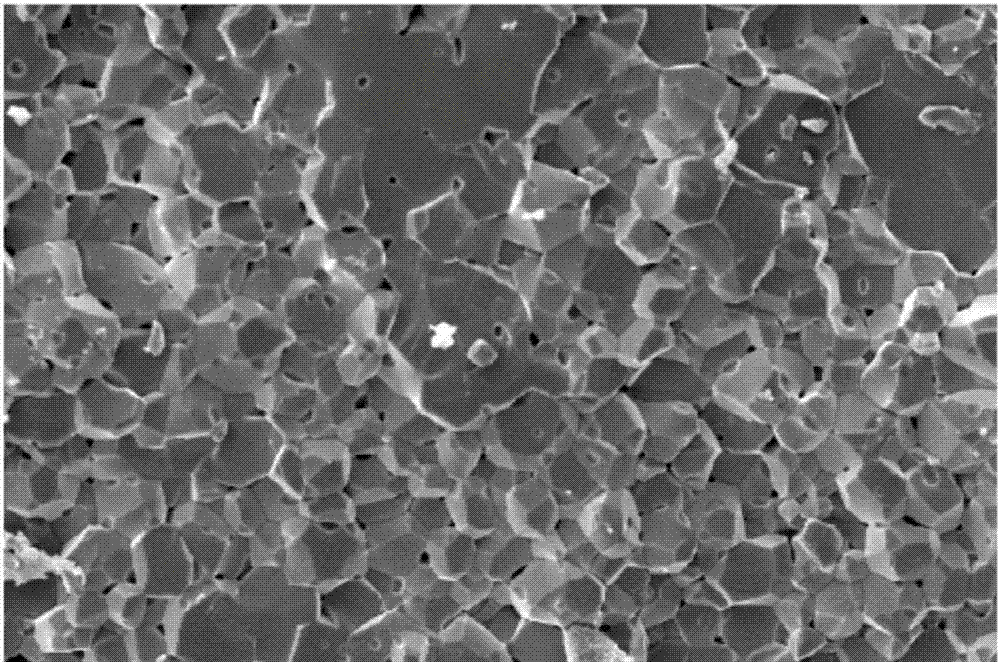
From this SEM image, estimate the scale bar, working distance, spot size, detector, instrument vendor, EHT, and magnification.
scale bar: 50000 nm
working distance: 9.4 mm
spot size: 5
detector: SE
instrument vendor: FEI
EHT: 15 kV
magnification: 0.499 K X